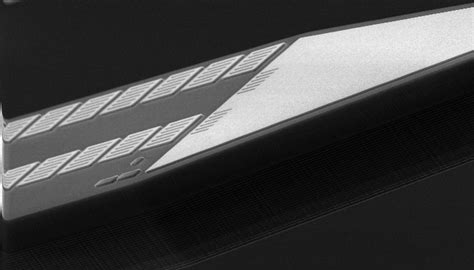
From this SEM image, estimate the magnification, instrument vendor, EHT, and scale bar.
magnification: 2.431 K X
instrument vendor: FEI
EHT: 5 kV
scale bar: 20000 nm